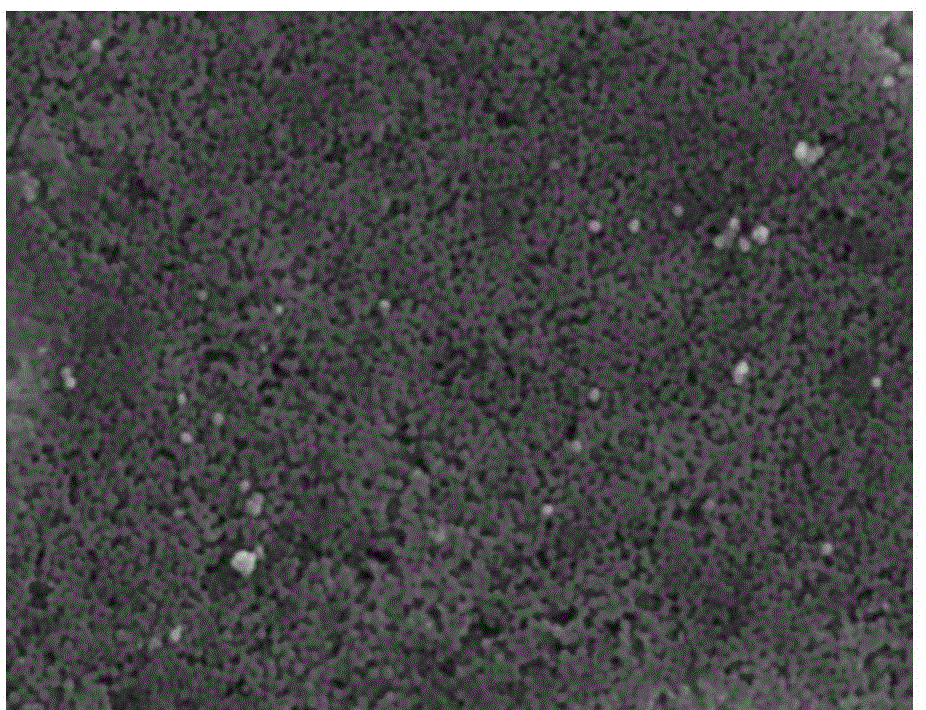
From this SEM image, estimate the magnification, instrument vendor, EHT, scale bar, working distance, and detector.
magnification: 3 K X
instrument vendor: Hitachi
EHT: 15 kV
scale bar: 10000 nm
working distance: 5.3 mm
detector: SE